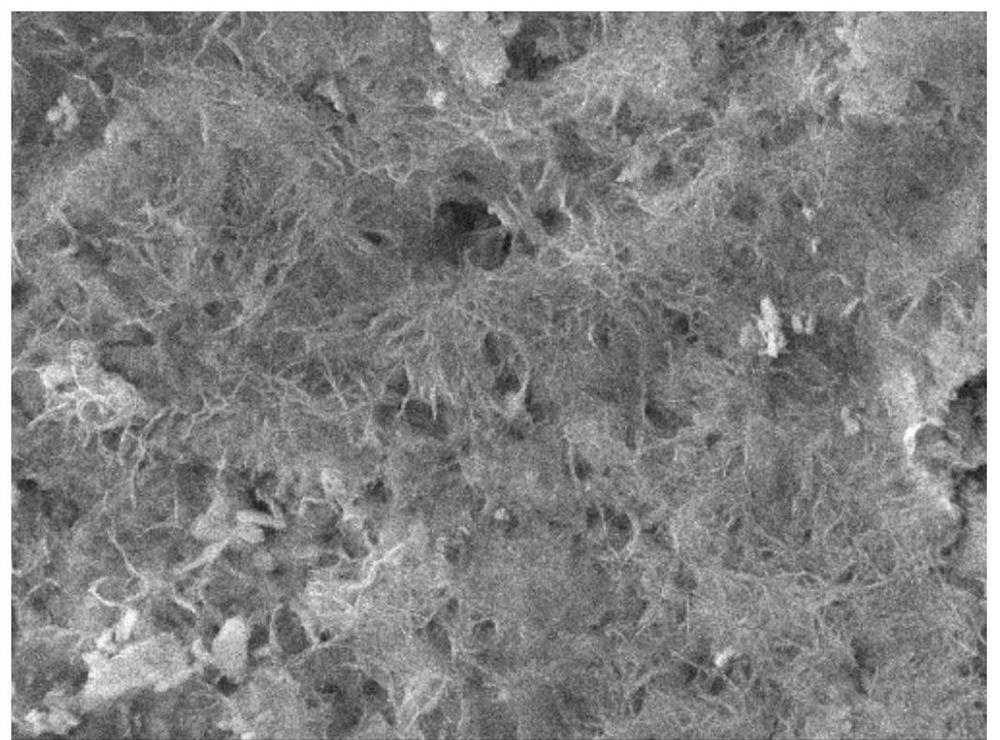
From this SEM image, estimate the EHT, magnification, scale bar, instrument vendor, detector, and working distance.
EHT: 8 kV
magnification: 10 K X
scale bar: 1000 nm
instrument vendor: JEOL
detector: SEI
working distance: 7.7 mm